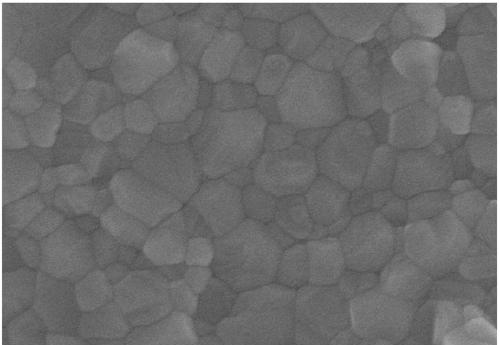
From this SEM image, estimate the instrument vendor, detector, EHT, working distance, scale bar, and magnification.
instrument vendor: Hitachi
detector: SE(U)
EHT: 10 kV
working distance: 8.7 mm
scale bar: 1000 nm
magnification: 50 K X